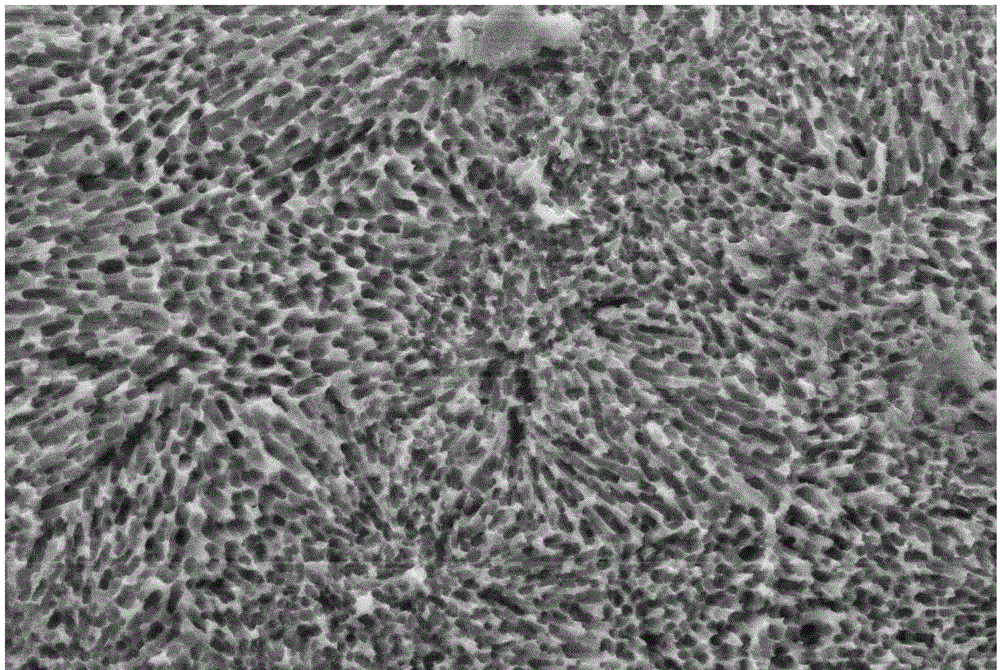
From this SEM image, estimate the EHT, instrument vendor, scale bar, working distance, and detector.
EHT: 15 kV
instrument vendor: Zeiss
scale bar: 2000 nm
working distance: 9 mm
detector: SE2